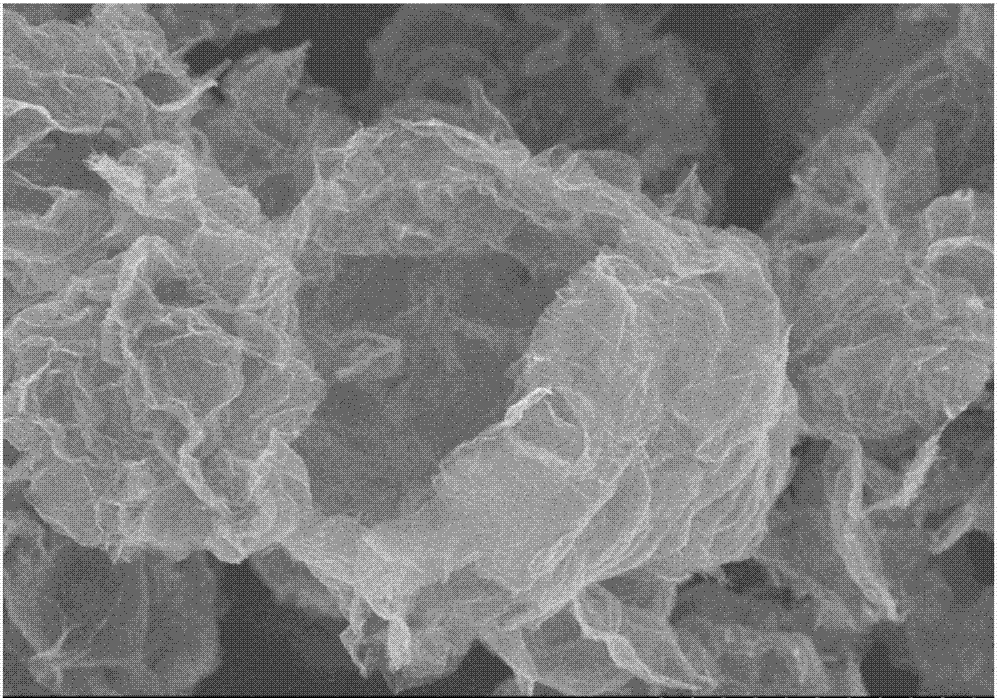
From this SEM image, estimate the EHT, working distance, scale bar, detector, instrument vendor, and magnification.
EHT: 3 kV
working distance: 11 mm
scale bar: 5000 nm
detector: SE(M)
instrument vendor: Hitachi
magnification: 8 K X